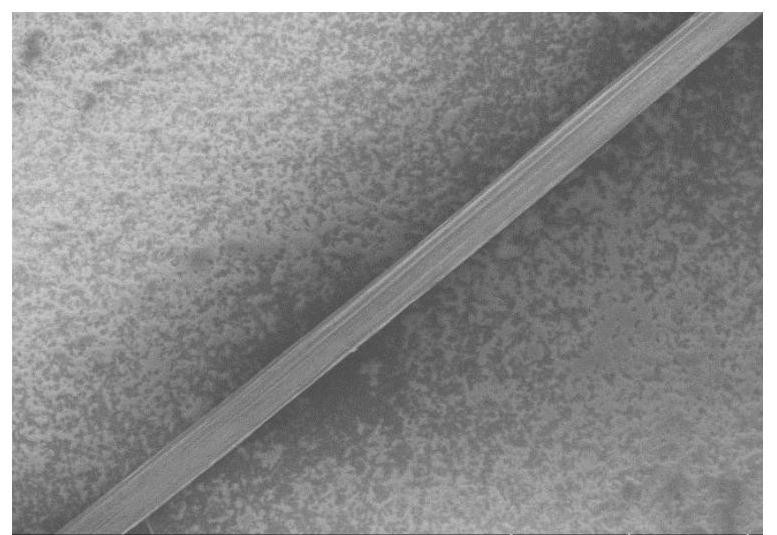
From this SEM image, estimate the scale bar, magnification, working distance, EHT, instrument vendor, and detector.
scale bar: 100000 nm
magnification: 0.4 K X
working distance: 8.5 mm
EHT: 2 kV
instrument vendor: Hitachi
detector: SE(U)